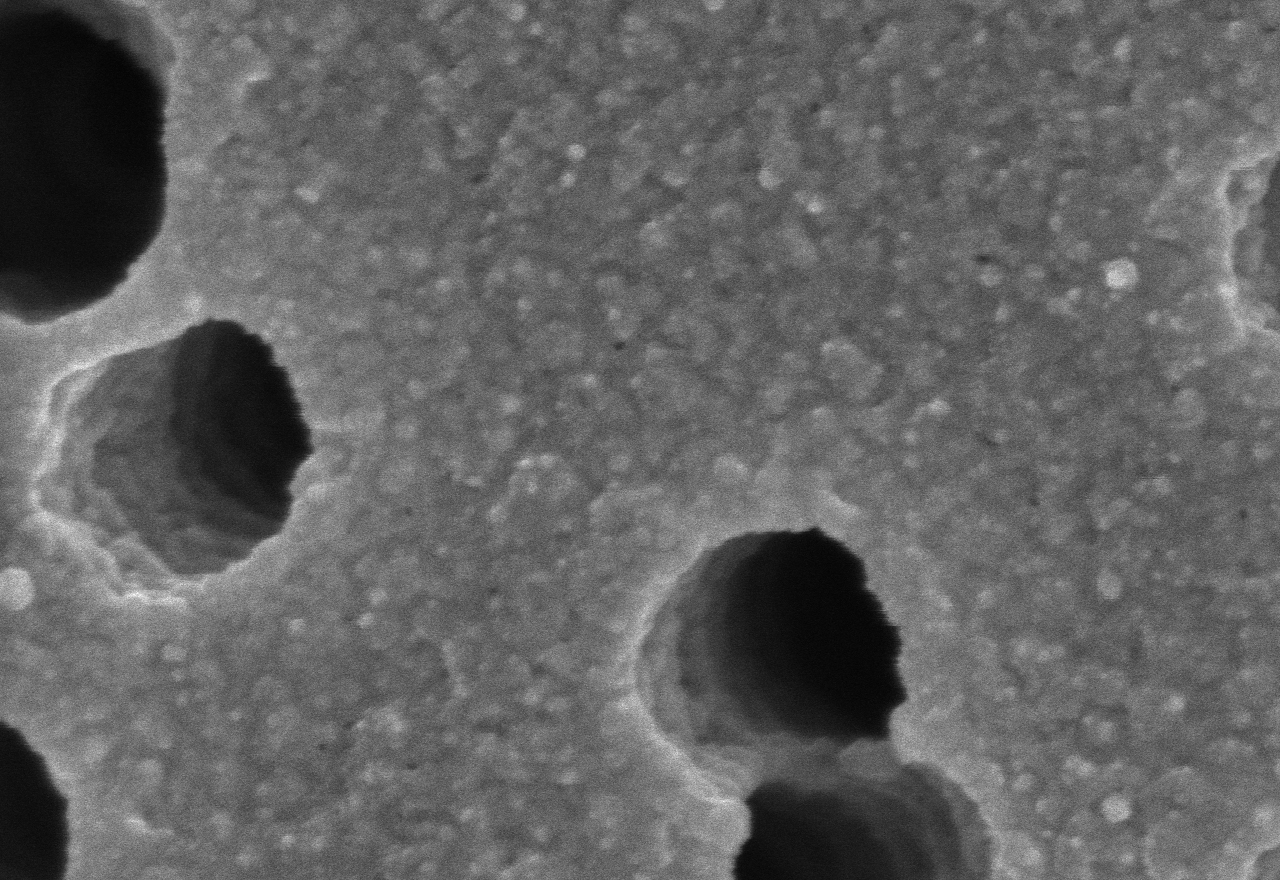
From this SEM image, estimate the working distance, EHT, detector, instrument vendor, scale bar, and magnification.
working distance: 4 mm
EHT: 3 kV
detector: SE(U)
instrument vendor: Hitachi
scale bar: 500 nm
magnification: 100 K X